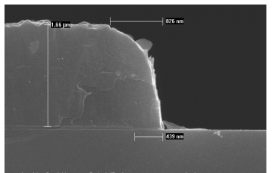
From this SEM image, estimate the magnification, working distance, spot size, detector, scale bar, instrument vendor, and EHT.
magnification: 30 K X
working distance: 5.2 mm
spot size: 3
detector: TLD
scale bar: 1000 nm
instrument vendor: FEI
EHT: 10 kV